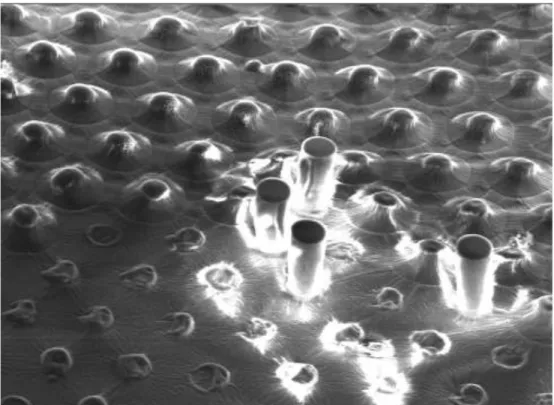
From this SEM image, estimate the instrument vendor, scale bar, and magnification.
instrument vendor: Tescan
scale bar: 100000 nm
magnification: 0.5 K X